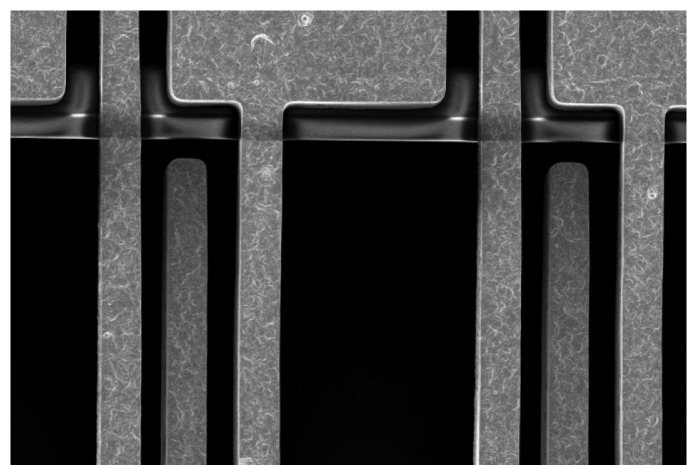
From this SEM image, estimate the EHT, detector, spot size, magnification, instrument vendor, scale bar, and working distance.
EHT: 10 kV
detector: SEI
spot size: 40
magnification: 0.9 K X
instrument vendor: JEOL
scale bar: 20000 nm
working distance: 20 mm